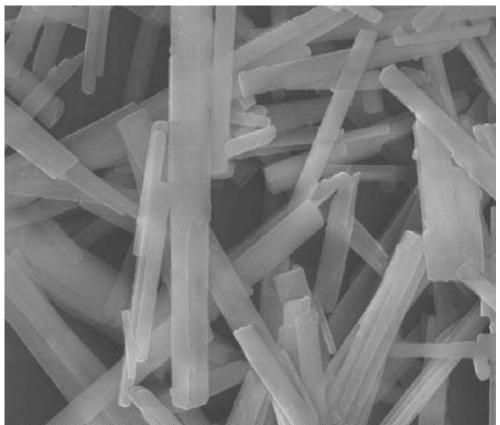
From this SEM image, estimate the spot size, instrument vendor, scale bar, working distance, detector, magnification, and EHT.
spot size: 3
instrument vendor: FEI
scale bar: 1000 nm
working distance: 6 mm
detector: TLD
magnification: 50 K X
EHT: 10 kV